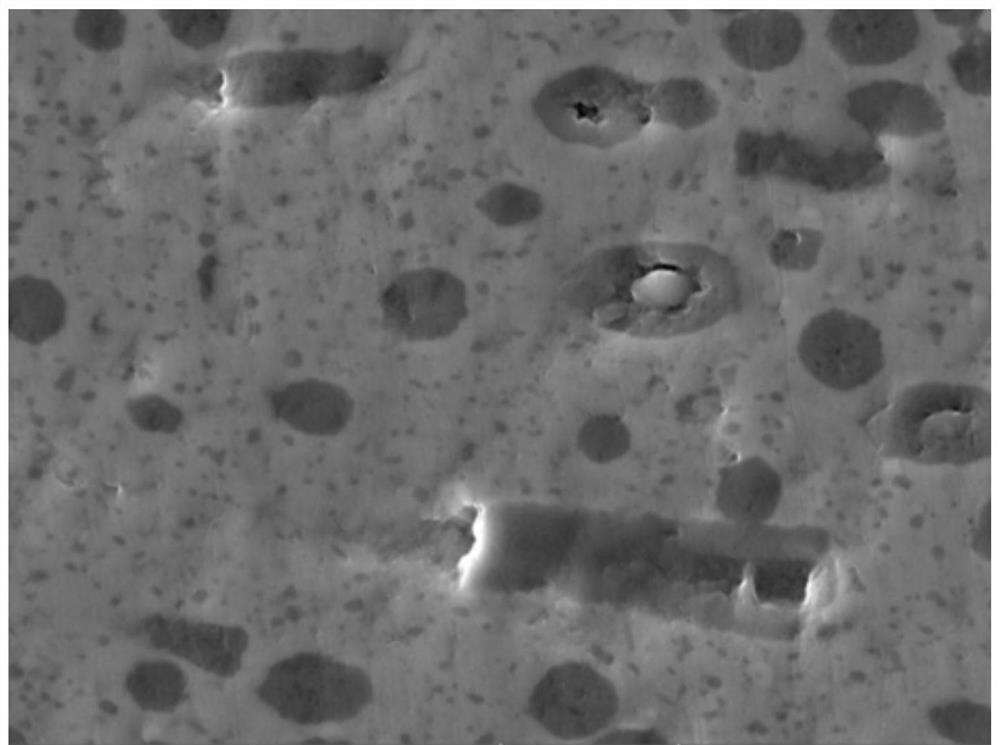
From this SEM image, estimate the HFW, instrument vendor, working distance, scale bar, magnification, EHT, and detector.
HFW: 55.4 µm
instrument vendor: Tescan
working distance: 9.98 mm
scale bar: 10000 nm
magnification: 5 K X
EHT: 20 kV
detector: SE